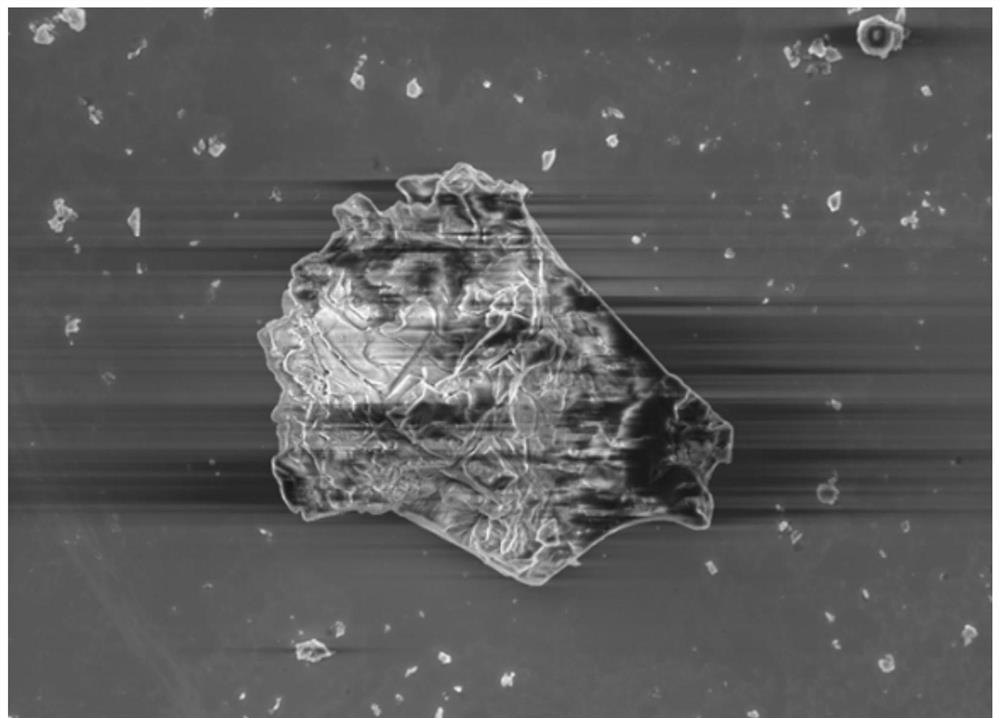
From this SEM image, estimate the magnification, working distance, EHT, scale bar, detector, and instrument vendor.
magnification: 2 K X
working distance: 8.8 mm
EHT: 5 kV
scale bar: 2000 nm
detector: InLens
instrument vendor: Zeiss